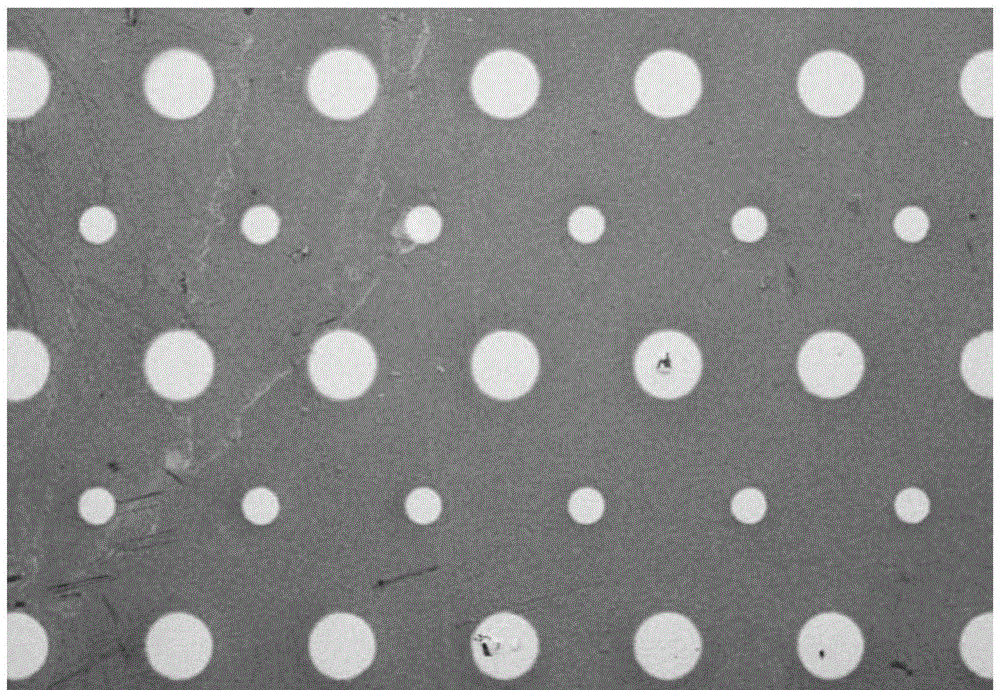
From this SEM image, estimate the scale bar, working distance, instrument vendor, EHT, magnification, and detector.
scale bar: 1000000 nm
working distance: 7.3 mm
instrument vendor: Hitachi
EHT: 3 kV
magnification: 0.035 K X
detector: SE(M)